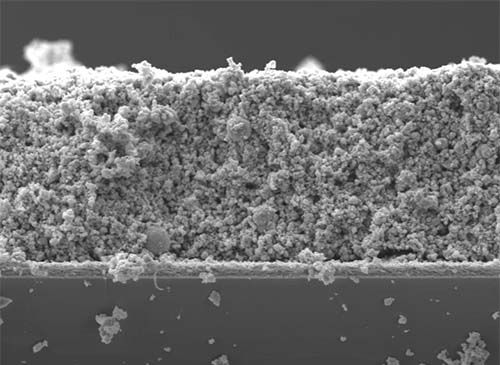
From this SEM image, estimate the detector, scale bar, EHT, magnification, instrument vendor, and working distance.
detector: LED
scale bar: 1000 nm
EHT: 5 kV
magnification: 5 K X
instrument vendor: JEOL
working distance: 10.4 mm